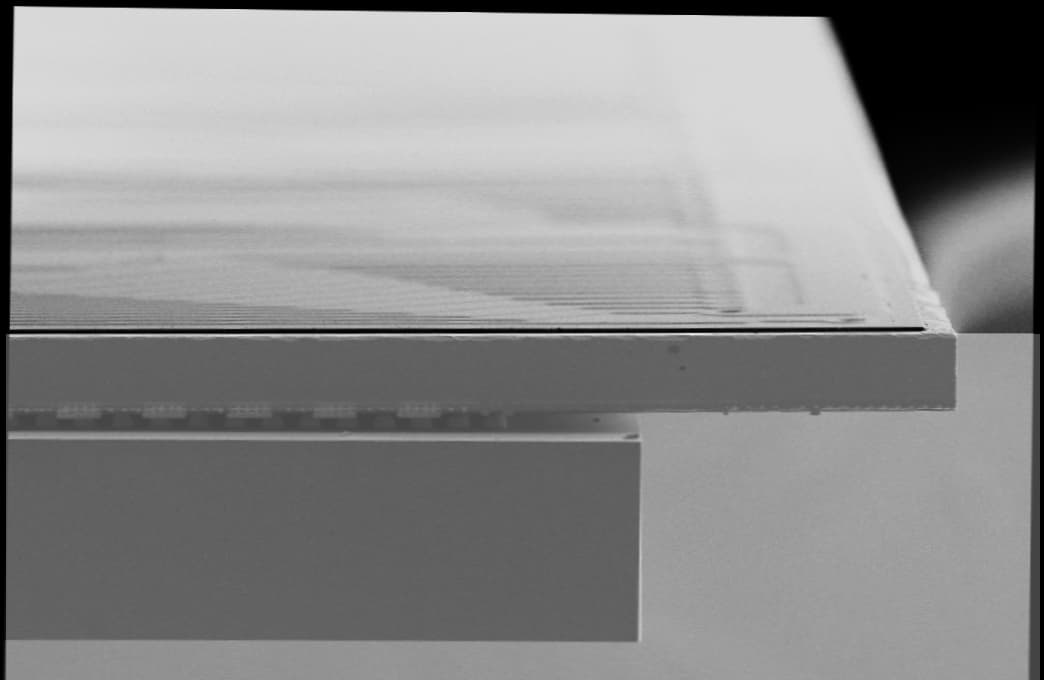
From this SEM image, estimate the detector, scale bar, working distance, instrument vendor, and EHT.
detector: SE2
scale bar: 100000 nm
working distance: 4 mm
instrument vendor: Zeiss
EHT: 5 kV